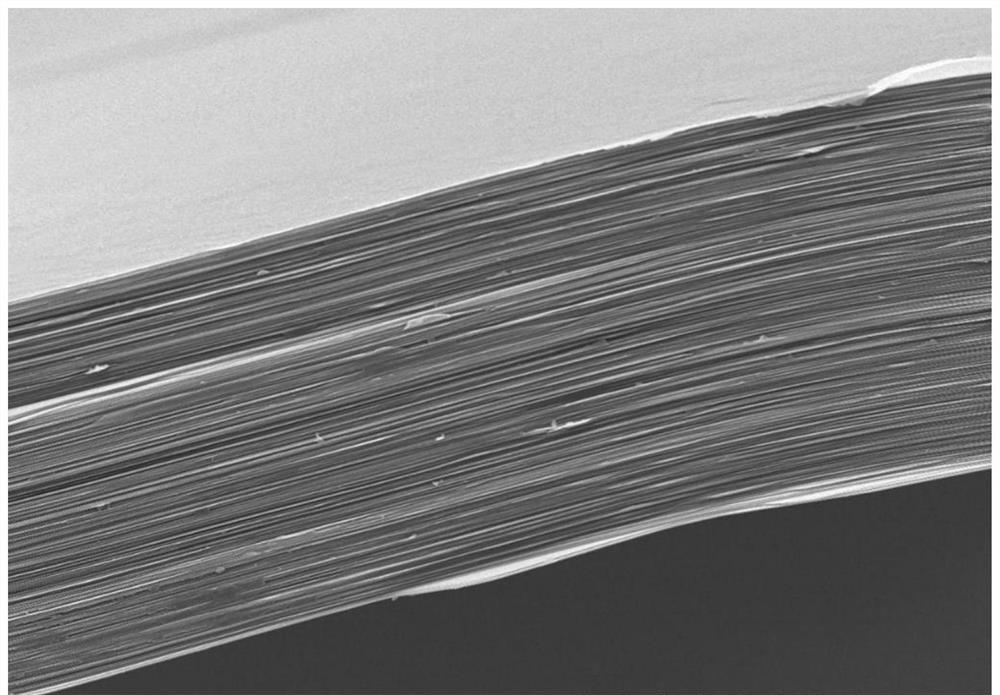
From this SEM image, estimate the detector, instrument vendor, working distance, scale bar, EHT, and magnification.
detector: SE(U)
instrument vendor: Hitachi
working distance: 9.1 mm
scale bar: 10000 nm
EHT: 10 kV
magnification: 5 K X